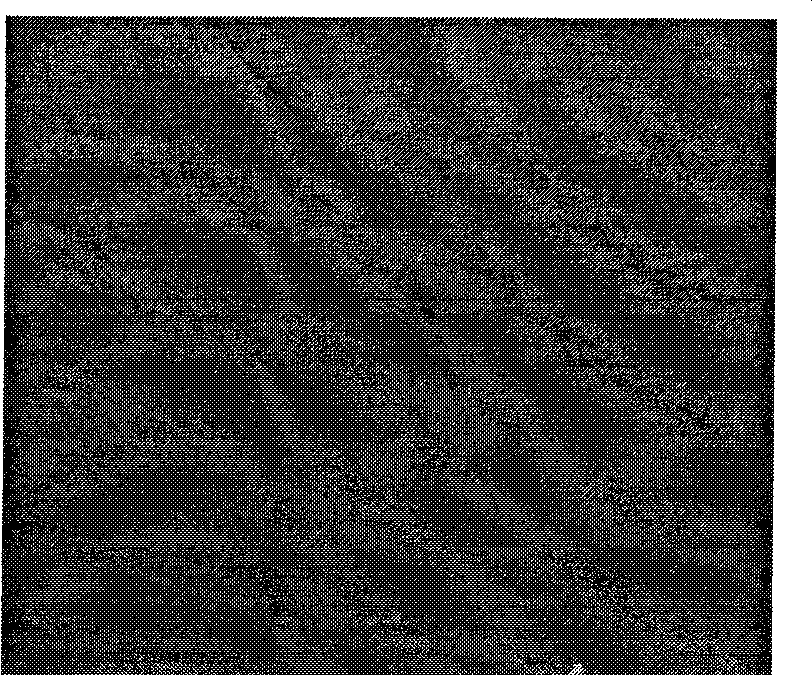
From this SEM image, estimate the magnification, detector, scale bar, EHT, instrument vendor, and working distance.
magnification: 0.05 K X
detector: SE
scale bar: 2000000 nm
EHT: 10 kV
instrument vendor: FEI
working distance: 12 mm